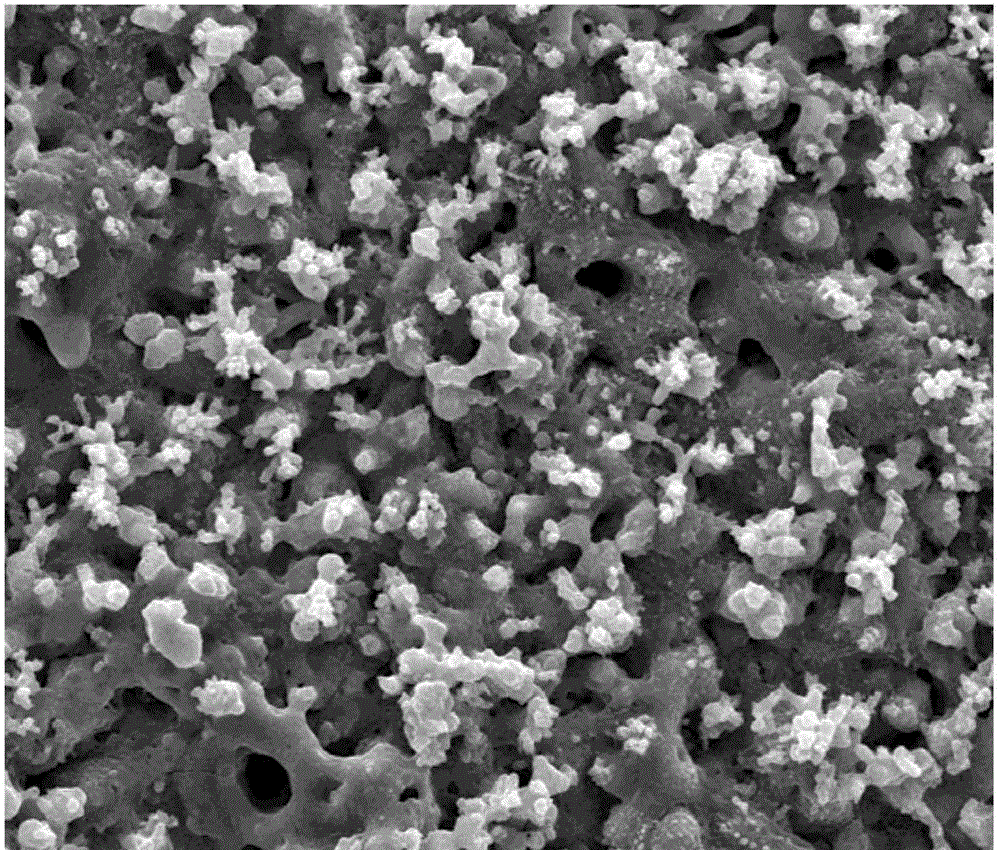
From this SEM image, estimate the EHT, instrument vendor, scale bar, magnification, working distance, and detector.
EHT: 30 kV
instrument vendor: FEI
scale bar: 50000 nm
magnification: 2 K X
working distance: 10.5 mm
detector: SE